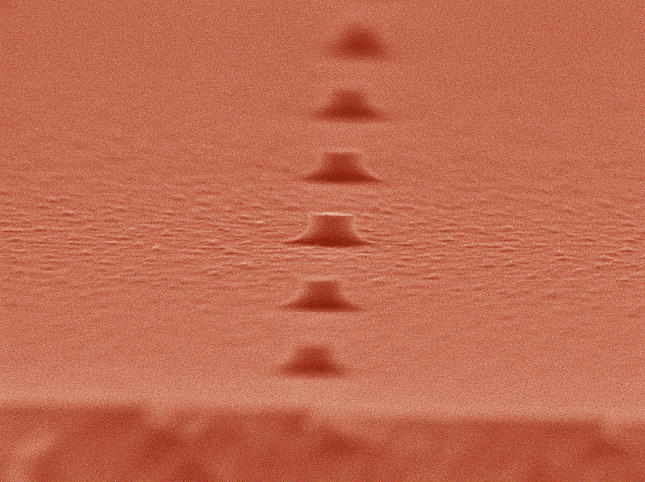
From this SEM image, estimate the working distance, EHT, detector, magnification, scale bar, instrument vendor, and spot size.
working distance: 5 mm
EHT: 20 kV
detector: TLD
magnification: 80 K X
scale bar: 200 nm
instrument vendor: FEI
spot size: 3